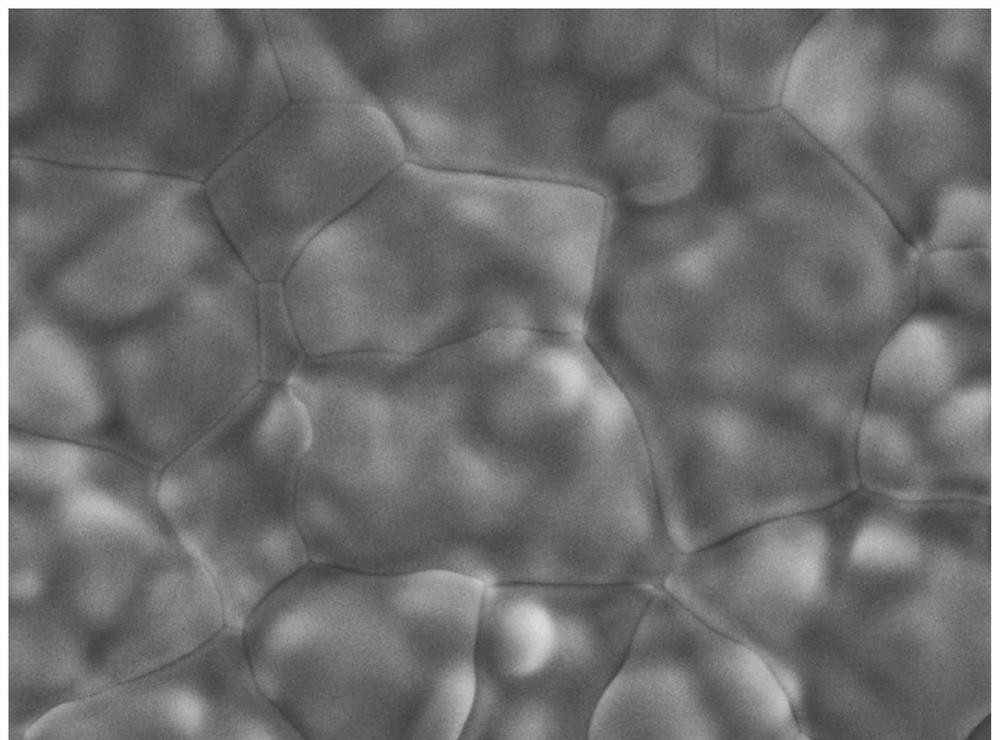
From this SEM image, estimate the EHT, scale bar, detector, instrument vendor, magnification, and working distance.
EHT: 15 kV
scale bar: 1000 nm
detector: SEI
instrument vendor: JEOL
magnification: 5 K X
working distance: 10 mm